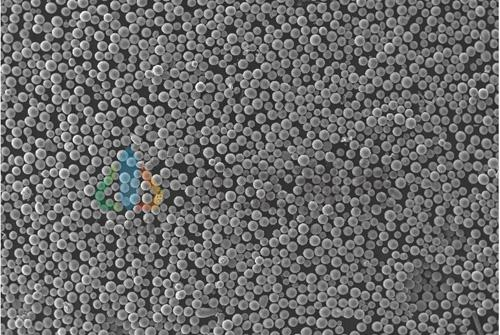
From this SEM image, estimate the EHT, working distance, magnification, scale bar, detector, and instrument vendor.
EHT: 30 kV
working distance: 5.47 mm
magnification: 0.1 K X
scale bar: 500000 nm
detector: SE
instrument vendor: Tescan